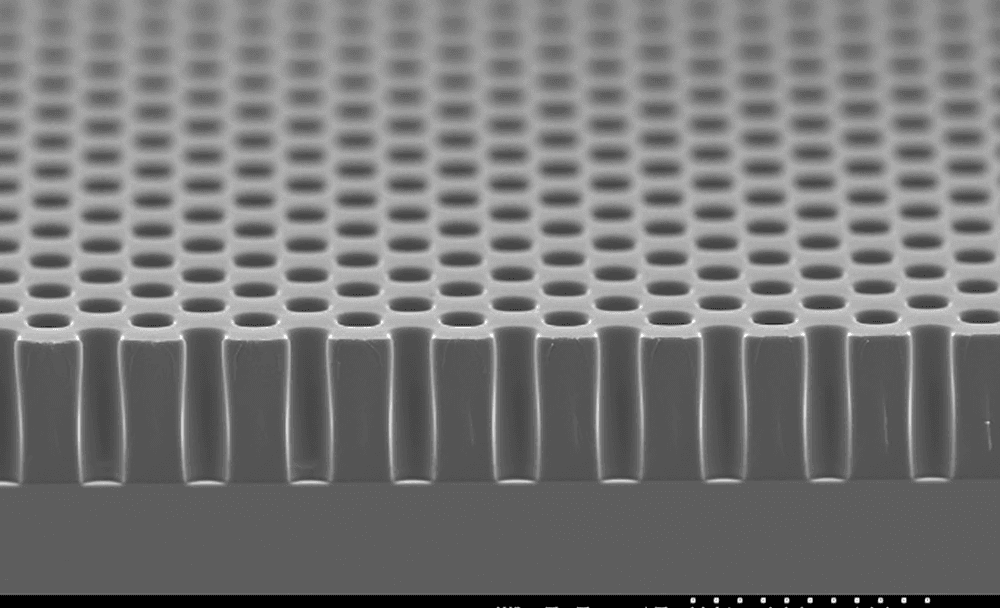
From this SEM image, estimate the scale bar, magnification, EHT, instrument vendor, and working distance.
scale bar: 100000 nm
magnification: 0.3 K X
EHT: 15 kV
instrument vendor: JEOL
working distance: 5.5 mm